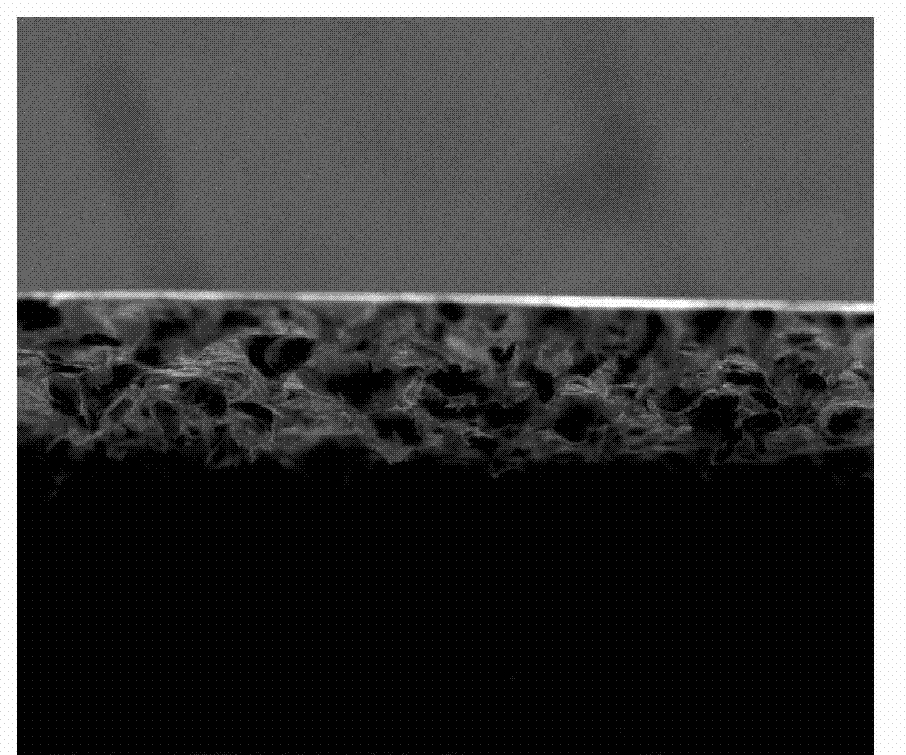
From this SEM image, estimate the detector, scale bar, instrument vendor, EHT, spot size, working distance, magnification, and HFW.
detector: ETD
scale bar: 300000 nm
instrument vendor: FEI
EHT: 20 kV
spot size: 3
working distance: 11.7 mm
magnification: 0.4 K X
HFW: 746 µm